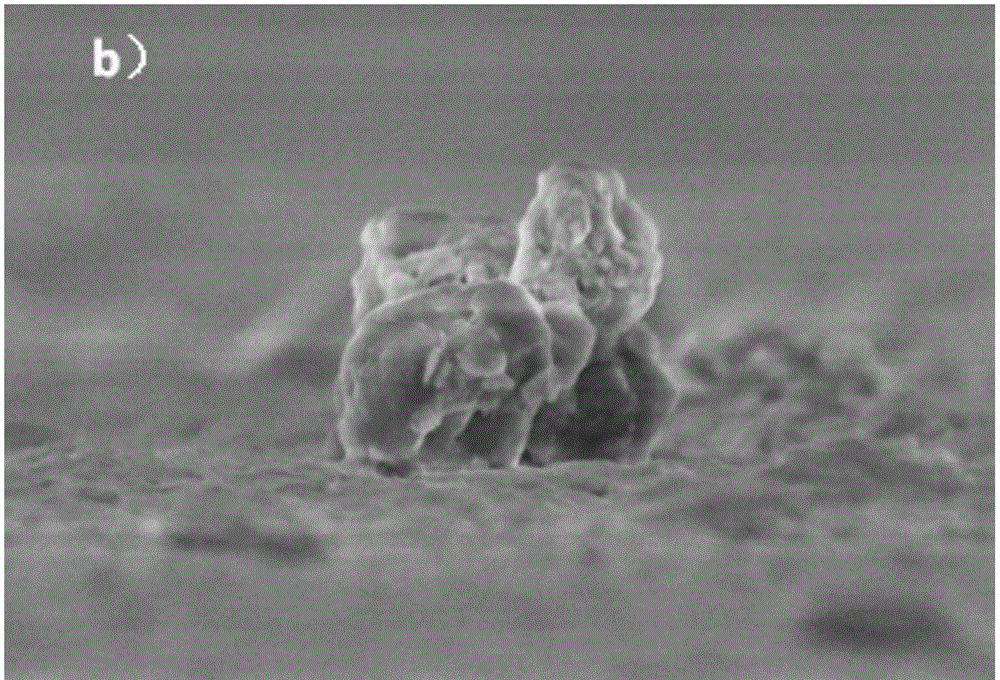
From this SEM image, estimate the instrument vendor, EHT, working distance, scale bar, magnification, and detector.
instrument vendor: Hitachi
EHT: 3 kV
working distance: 9.1 mm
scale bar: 2000 nm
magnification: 20 K X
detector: SE(UL)